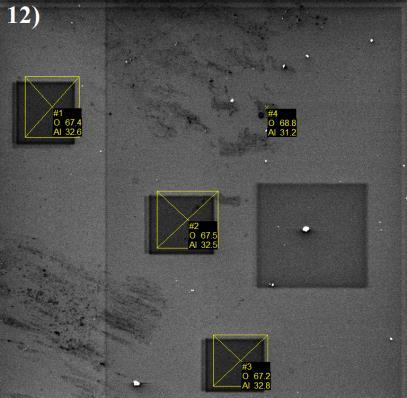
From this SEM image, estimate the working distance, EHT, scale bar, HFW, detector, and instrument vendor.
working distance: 15.76 mm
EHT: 5 kV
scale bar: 50000 nm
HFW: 215 µm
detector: SE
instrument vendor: Tescan